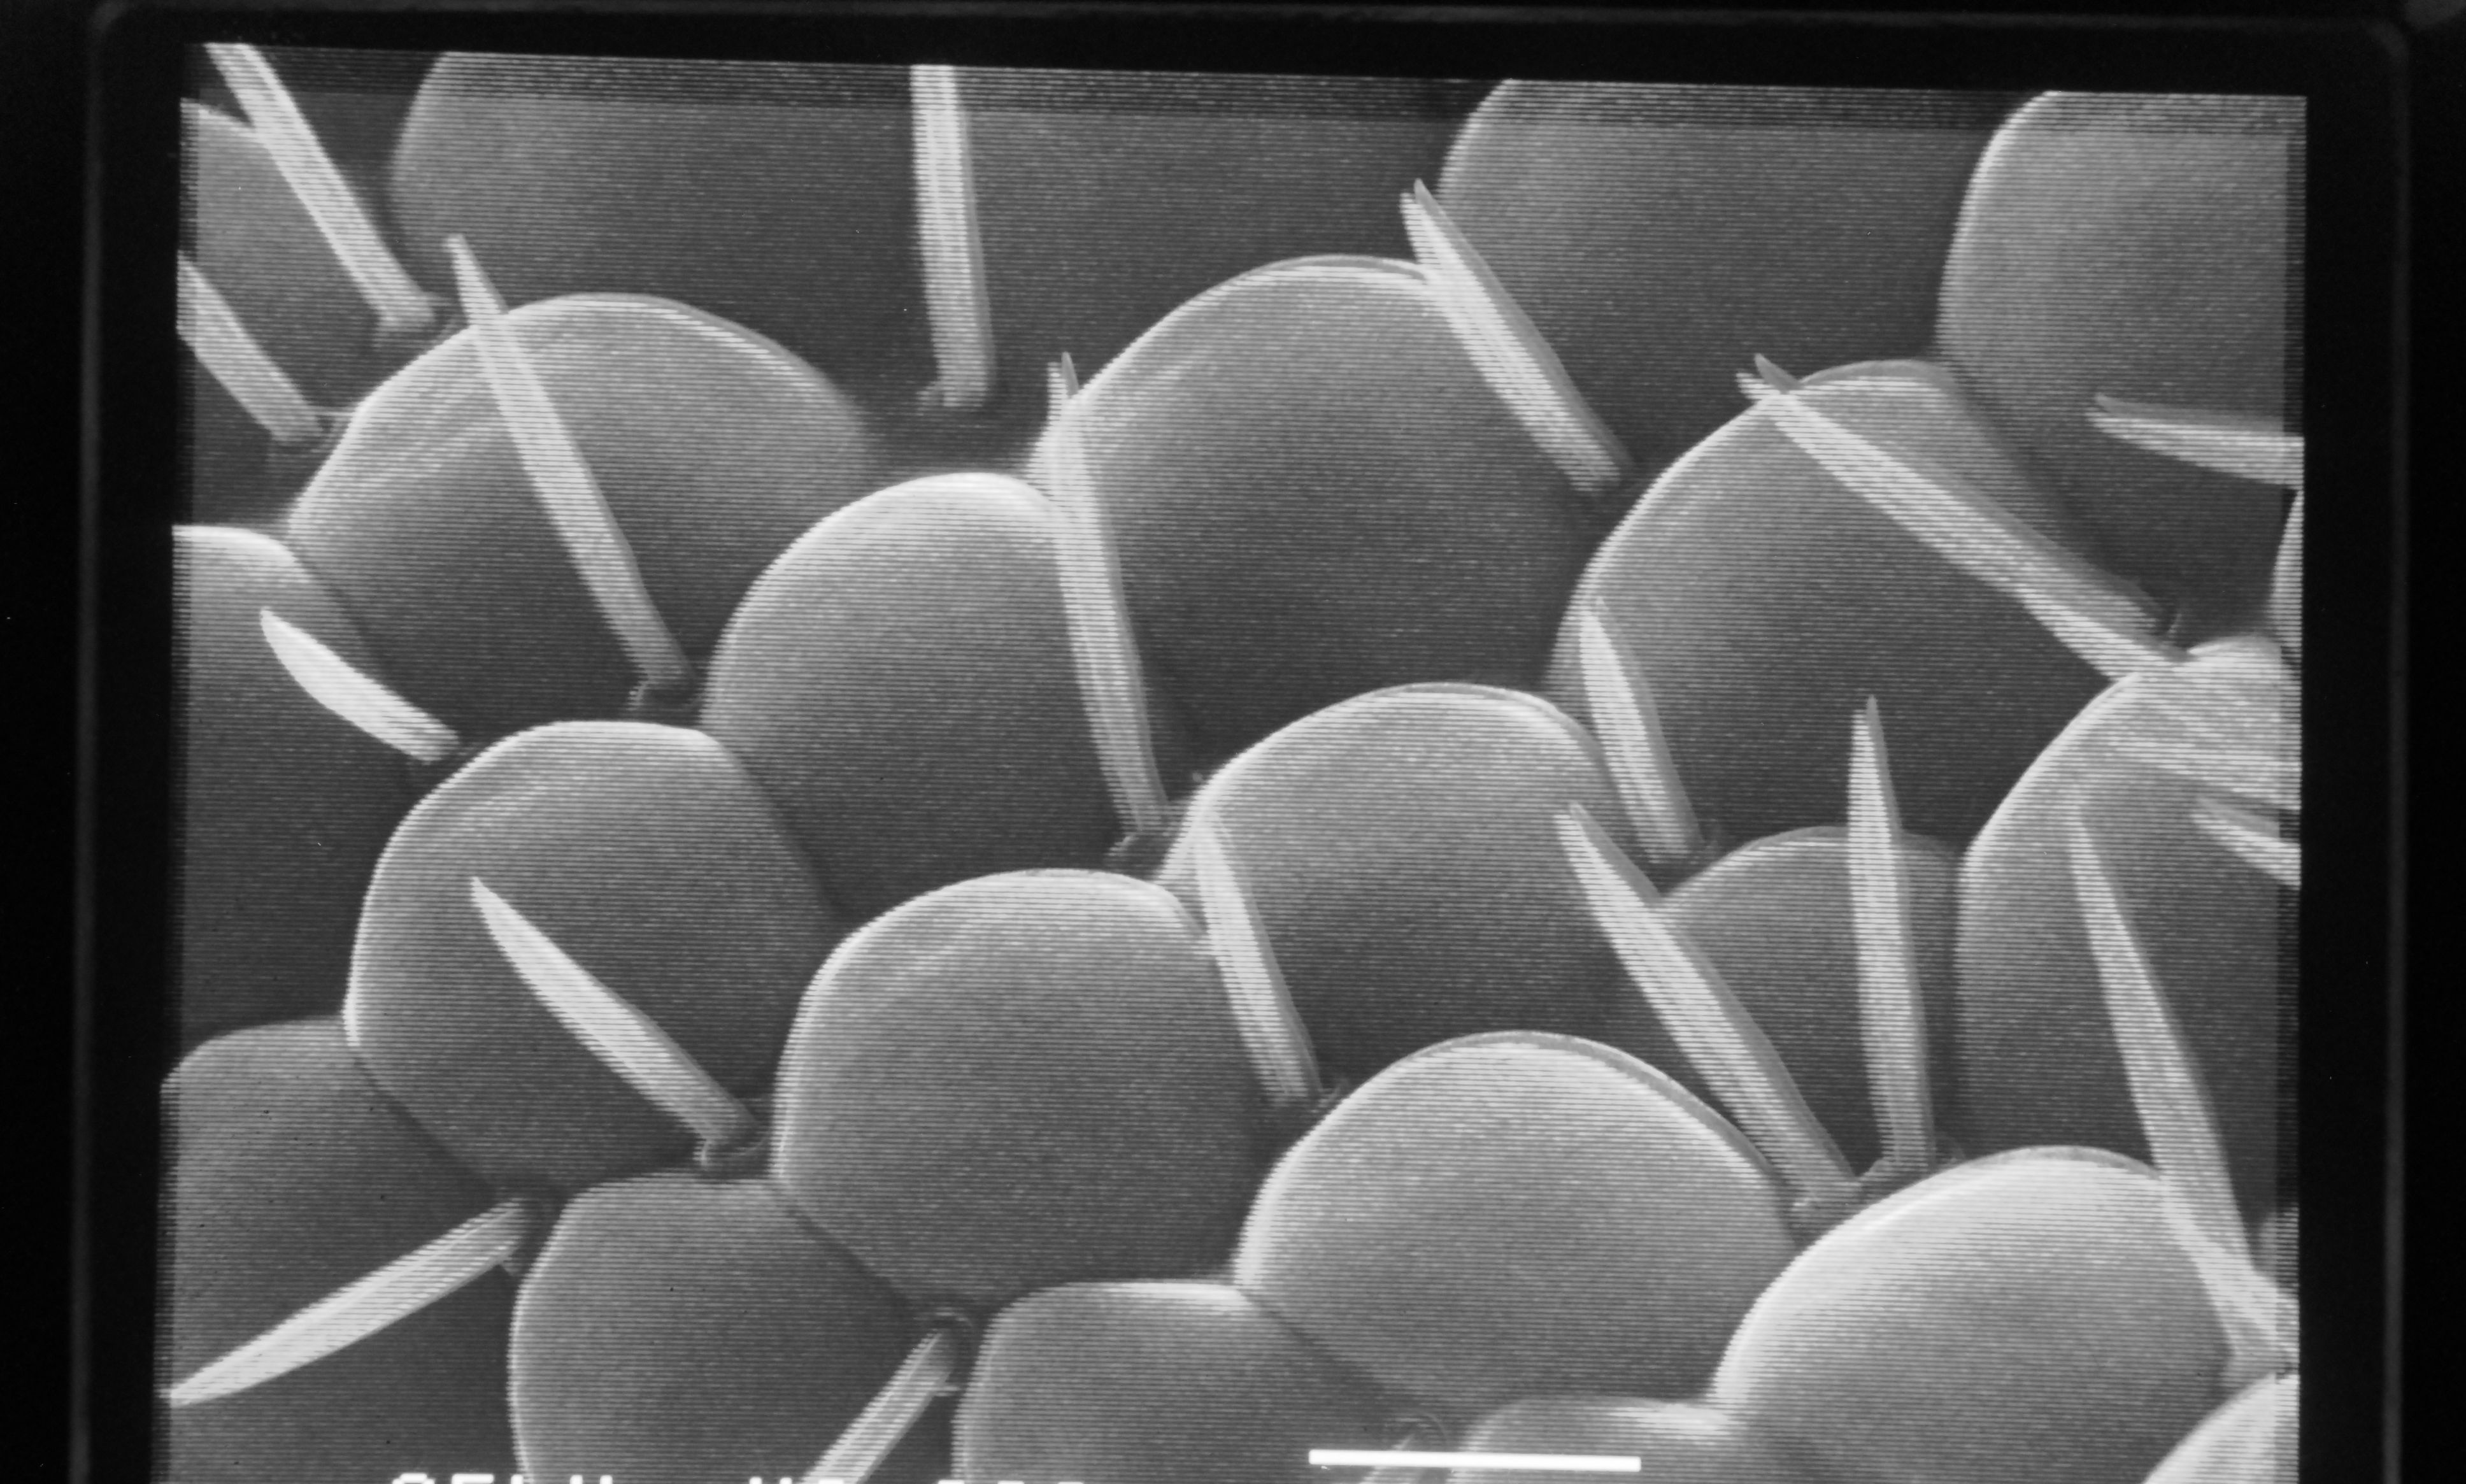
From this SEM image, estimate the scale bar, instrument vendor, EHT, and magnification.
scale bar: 10000 nm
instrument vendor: JEOL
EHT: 25 kV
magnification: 2 K X